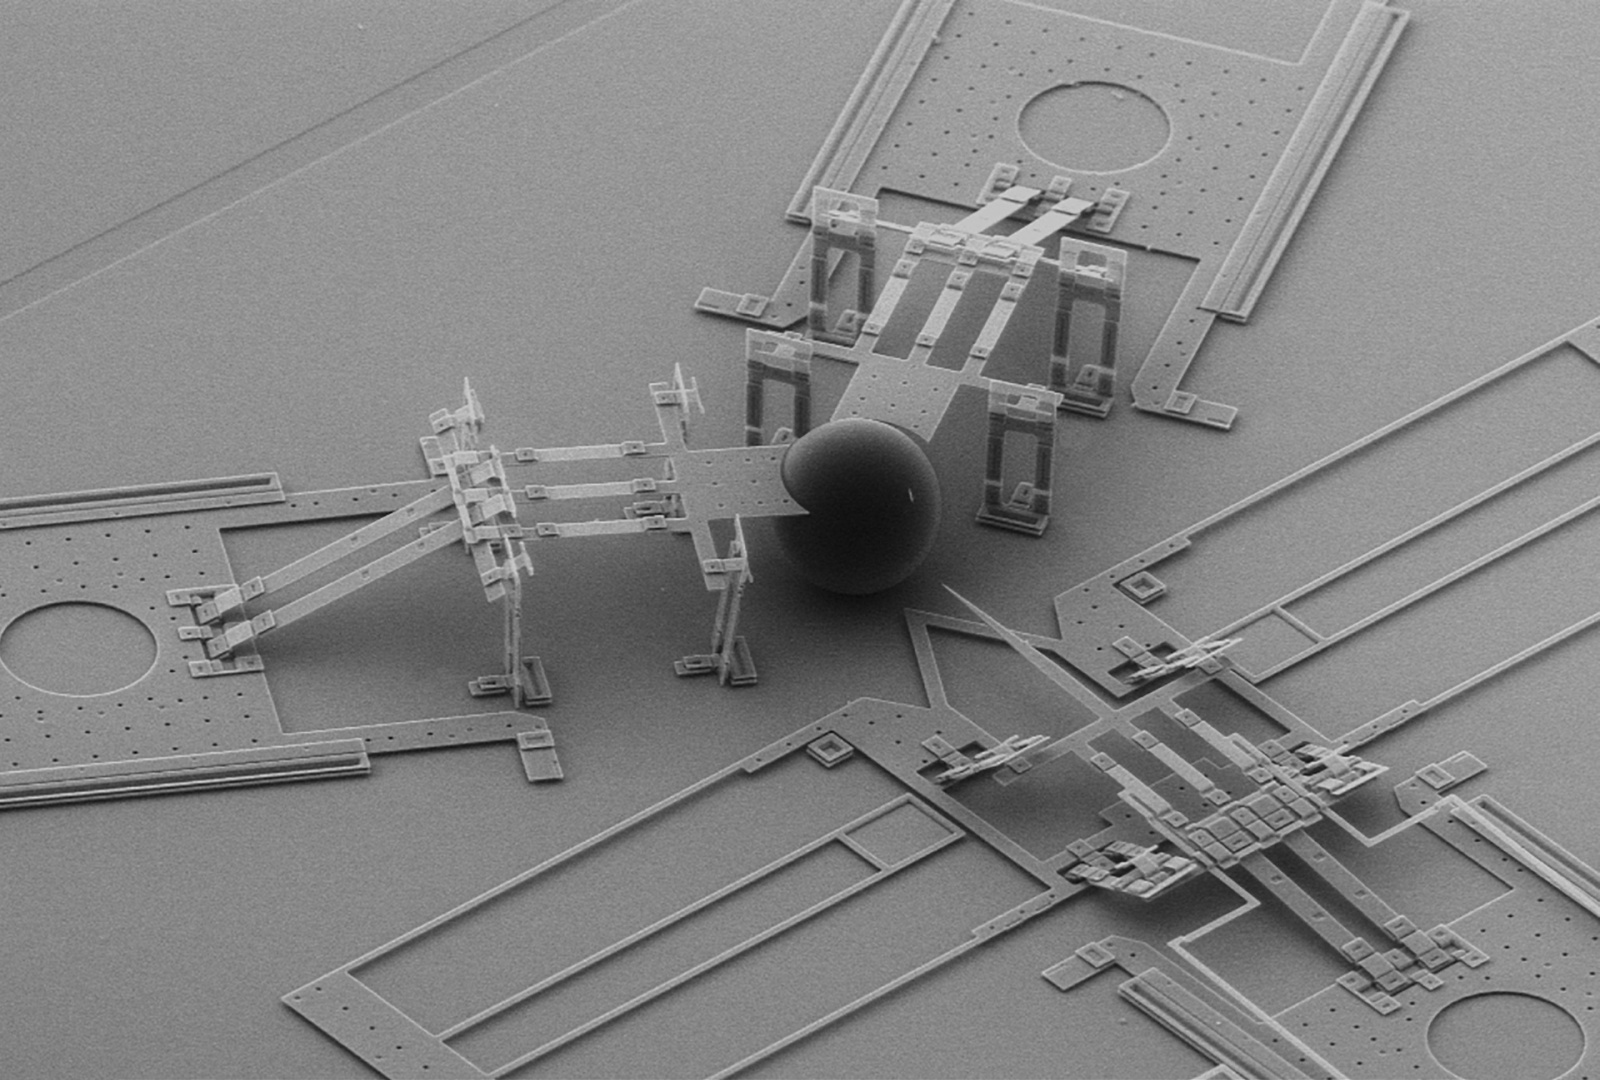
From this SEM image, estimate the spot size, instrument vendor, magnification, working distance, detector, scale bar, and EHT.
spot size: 3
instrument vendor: FEI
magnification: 0.137 K X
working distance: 12.7 mm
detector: GSE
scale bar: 200000 nm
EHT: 15 kV